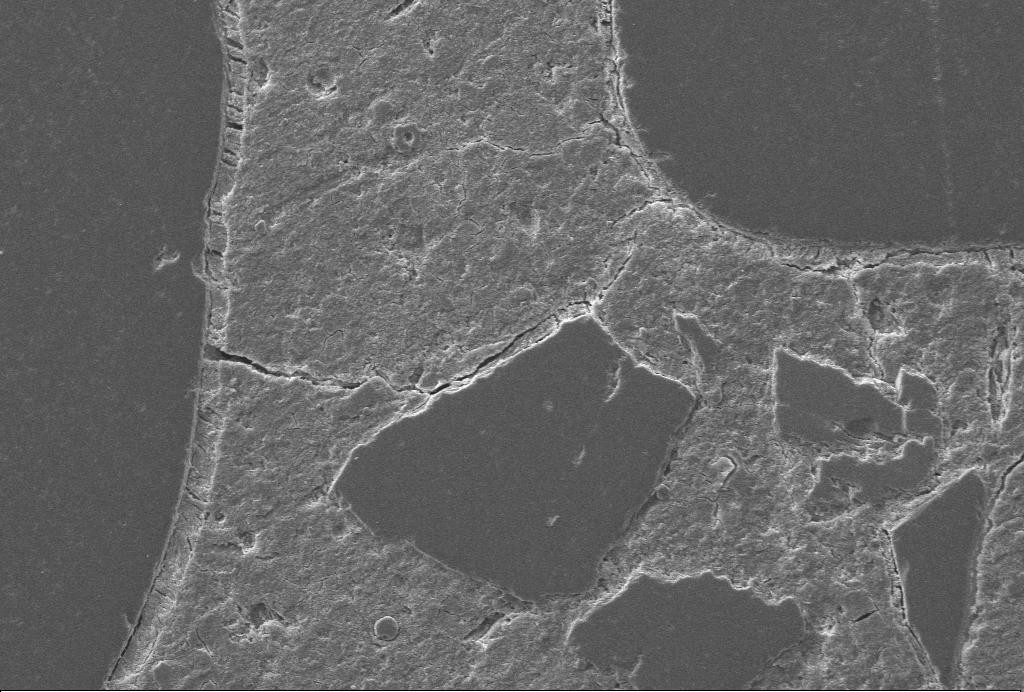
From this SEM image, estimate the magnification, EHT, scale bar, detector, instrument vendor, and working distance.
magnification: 0.461 K X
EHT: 15 kV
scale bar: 100000 nm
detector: SE1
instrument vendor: Zeiss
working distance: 13.5 mm